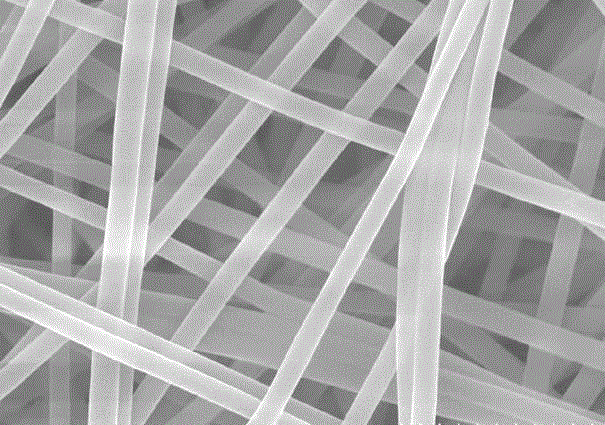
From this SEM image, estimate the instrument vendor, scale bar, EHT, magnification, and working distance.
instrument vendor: Hitachi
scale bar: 2000 nm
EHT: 15 kV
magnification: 20 K X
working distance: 11.3 mm